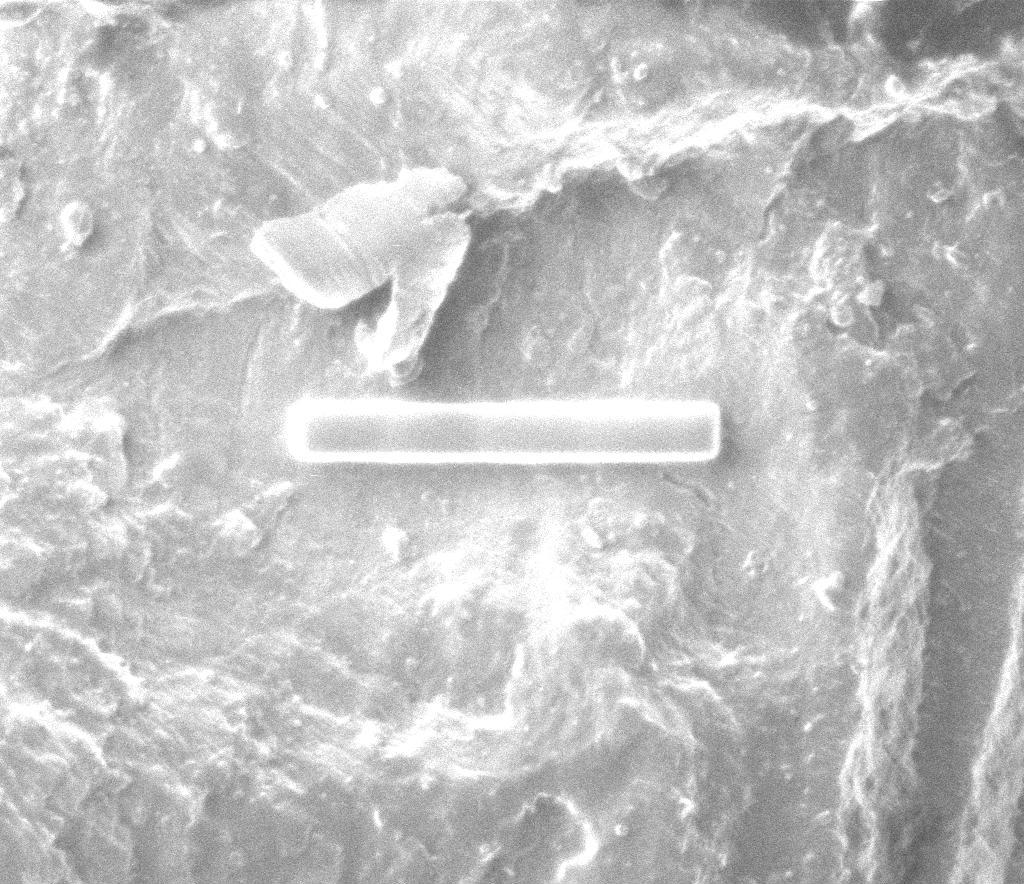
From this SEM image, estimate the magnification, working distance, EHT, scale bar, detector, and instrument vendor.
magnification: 3 K X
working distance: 19 mm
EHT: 30 kV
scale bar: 10000 nm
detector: CDEM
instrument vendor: FEI